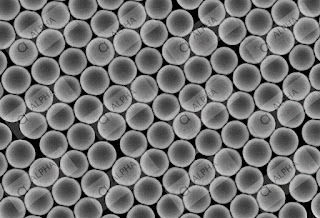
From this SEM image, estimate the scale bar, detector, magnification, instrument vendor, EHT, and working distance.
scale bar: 5000 nm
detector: SE(TUL)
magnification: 10 K X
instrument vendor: Hitachi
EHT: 3 kV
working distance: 7.5 mm